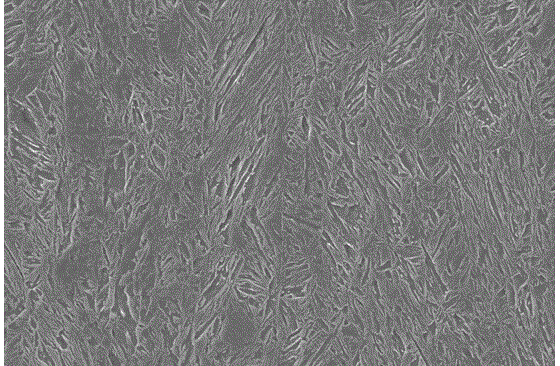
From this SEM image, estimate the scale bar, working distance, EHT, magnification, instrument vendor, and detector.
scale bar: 10000 nm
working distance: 17 mm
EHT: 20 kV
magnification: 1.5 K X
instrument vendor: Zeiss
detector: SE1